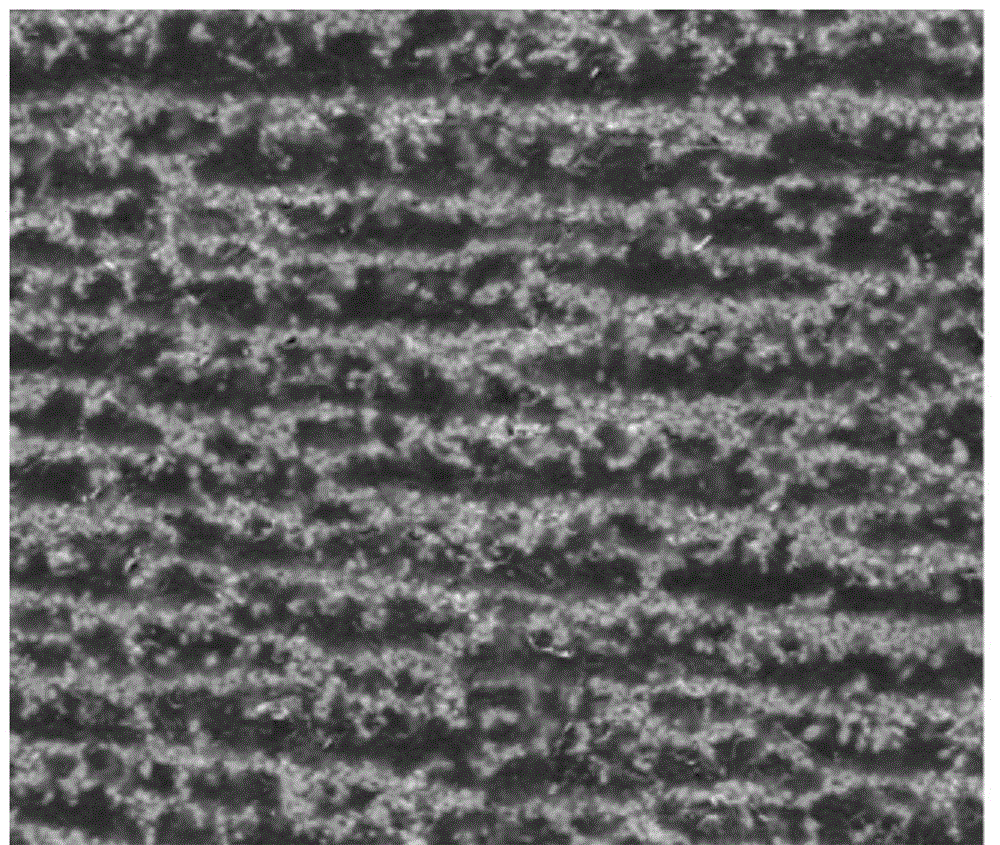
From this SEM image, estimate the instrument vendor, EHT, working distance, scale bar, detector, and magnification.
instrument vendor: FEI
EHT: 30 kV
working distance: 10.2 mm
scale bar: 50000 nm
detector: SE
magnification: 2 K X